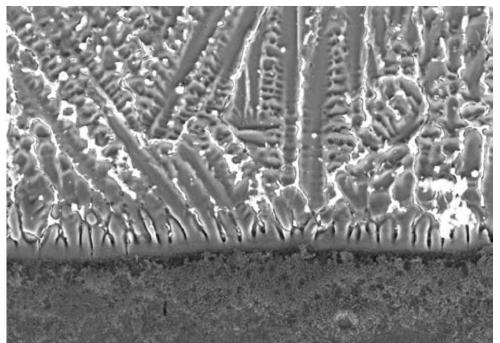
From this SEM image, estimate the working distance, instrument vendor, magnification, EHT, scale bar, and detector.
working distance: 10.5 mm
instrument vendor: Hitachi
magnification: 1 K X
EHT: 25 kV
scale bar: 50000 nm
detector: SE